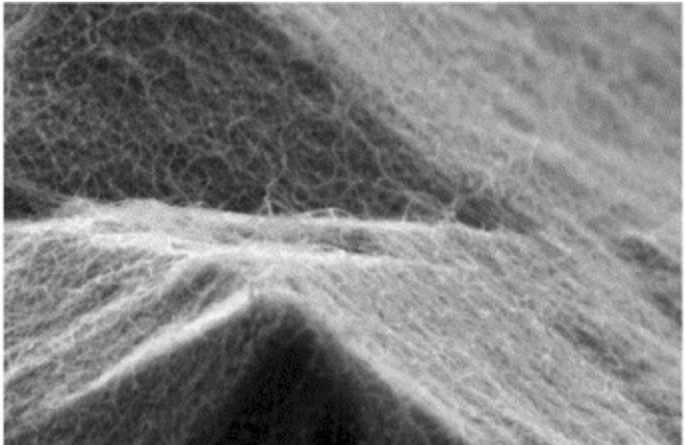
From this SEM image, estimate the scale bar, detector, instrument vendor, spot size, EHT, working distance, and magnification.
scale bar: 1000 nm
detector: SE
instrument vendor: FEI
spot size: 3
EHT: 5 kV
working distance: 11 mm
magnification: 50 K X